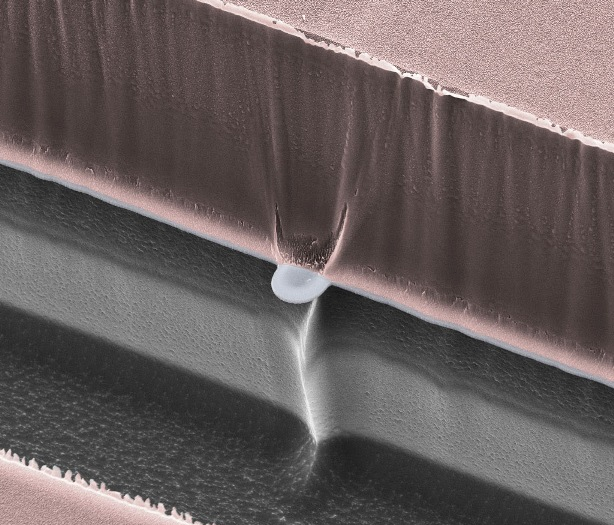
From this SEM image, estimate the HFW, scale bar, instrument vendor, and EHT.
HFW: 59.9 µm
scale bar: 20000 nm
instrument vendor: FEI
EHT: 15 kV